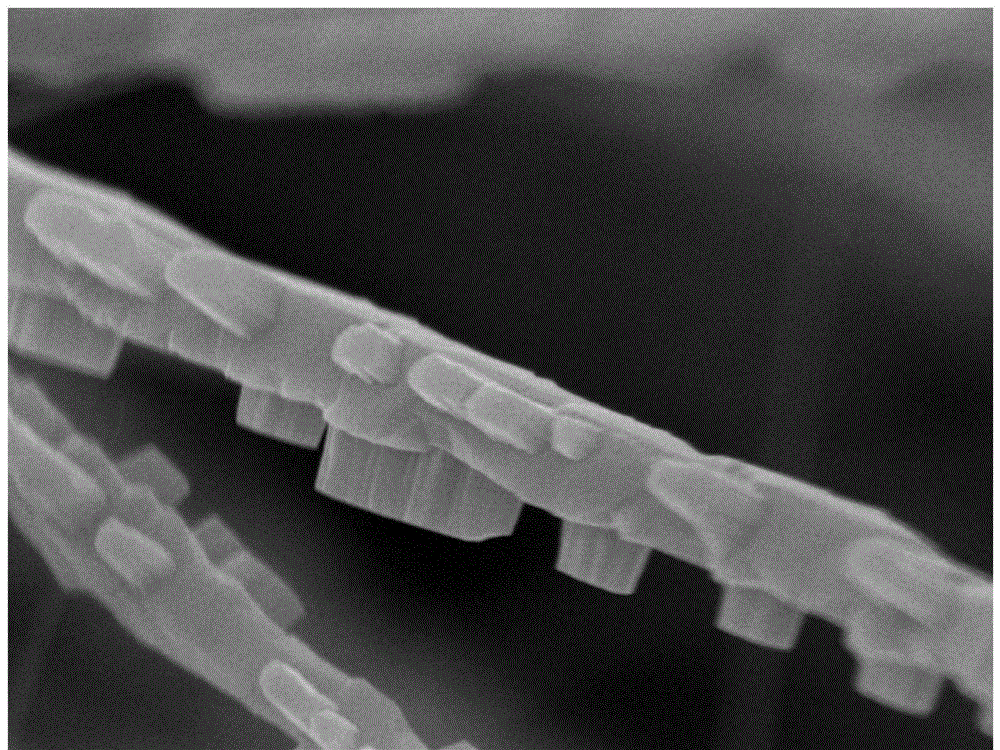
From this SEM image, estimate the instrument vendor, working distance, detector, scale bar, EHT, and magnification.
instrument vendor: JEOL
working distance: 2.8 mm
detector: SEI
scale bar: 100 nm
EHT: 10 kV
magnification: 33 K X